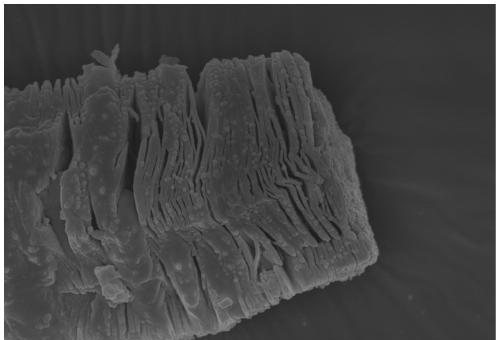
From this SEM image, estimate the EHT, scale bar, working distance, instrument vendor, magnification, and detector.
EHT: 10 kV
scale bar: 1000 nm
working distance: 10.3 mm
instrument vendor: Zeiss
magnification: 5 K X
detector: InLens